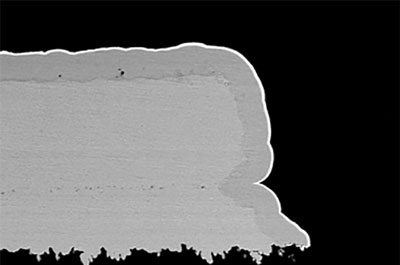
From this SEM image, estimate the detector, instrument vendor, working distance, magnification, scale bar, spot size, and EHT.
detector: BEC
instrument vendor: JEOL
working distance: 7 mm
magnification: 1.3 K X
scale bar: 10000 nm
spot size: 50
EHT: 15 kV